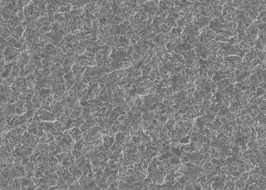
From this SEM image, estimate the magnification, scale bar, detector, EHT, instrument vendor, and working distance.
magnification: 0.5 K X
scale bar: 50000 nm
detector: SED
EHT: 10 kV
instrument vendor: JEOL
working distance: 11.6 mm